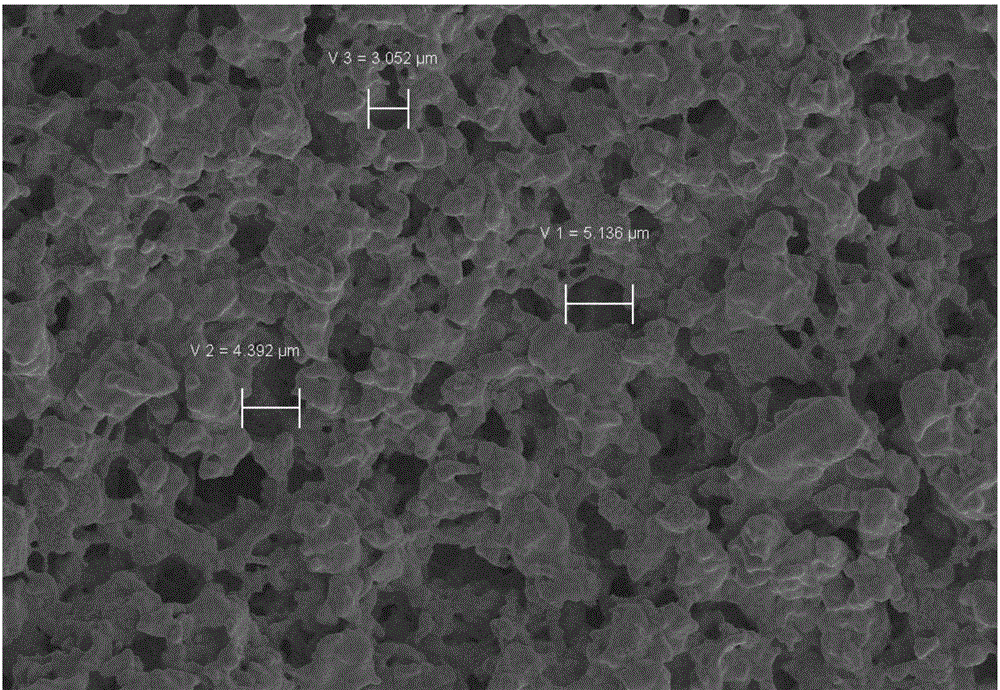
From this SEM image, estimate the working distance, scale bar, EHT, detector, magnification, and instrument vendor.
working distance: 13.5 mm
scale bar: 2000 nm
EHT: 5 kV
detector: SE2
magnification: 1.5 K X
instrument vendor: Zeiss